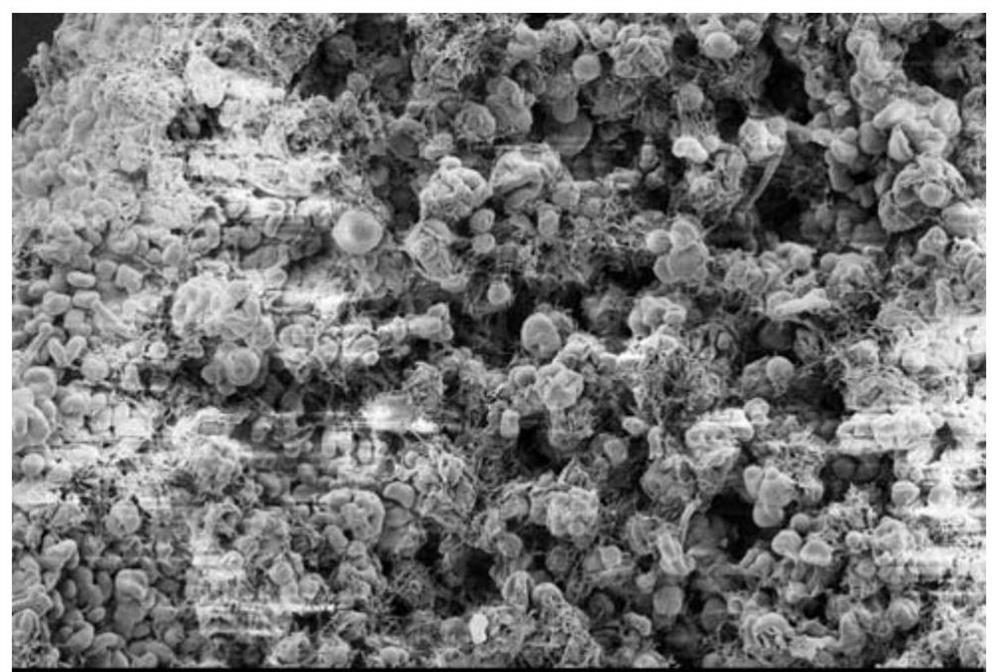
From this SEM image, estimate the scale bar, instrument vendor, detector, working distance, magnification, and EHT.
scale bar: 2000 nm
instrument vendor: Zeiss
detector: SE2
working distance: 5.3 mm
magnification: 2 K X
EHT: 1 kV